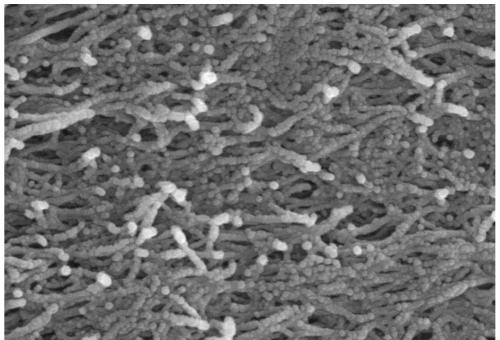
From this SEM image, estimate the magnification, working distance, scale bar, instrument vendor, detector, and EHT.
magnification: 70 K X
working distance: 8 mm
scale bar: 500 nm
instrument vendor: Hitachi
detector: SE(M)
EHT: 10 kV